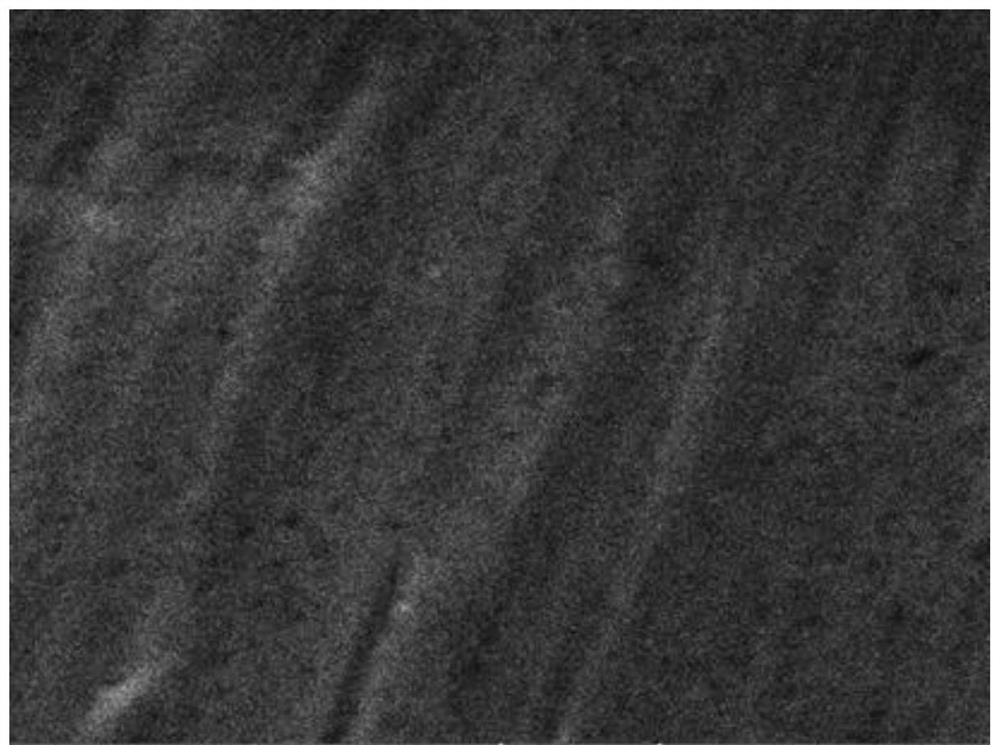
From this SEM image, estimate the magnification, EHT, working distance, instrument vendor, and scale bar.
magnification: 4.86 K X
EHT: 25 kV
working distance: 15 mm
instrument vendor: Tescan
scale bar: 5000 nm